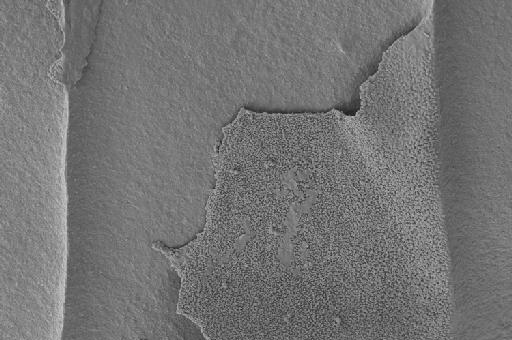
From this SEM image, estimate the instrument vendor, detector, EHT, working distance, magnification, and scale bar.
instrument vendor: Zeiss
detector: SE2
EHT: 4 kV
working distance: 5.9 mm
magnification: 4.31 K X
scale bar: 10000 nm